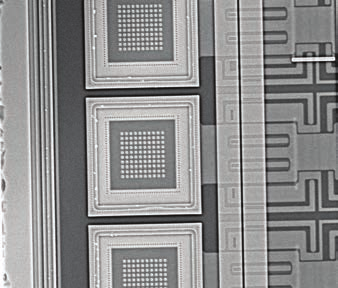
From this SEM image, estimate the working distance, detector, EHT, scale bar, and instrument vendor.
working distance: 9 mm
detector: NTS BSD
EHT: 22.78 kV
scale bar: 20000 nm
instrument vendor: Zeiss